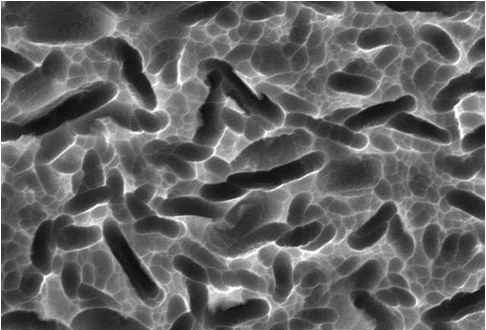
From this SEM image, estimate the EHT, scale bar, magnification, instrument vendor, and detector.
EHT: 20 kV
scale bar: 5000 nm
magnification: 3 K X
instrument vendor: JEOL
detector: SEI